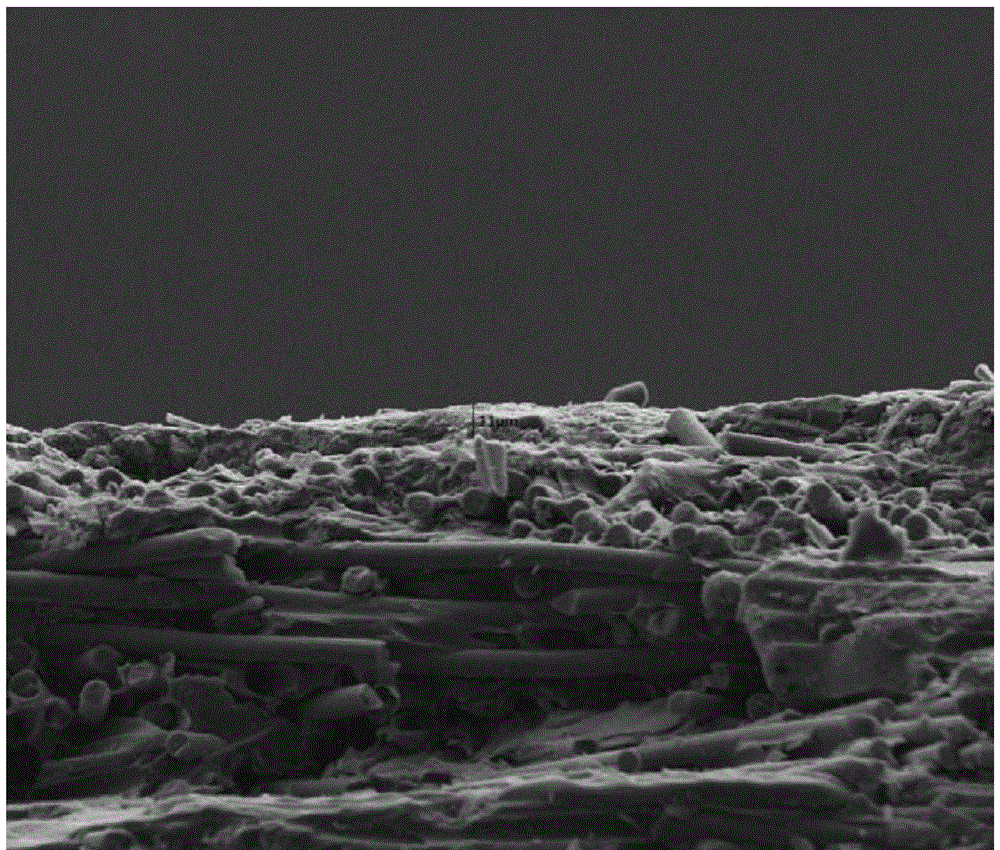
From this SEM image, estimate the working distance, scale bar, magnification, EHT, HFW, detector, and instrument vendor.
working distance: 5.1 mm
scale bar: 50000 nm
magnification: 1 K X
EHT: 10 kV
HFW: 240 µm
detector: ETD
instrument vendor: FEI